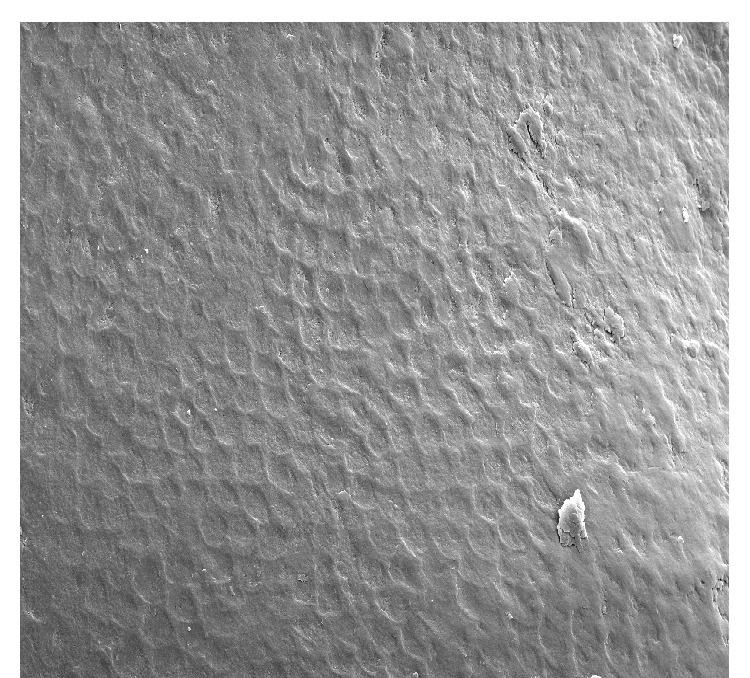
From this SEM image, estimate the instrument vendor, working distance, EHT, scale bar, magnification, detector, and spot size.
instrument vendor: FEI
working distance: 14.9 mm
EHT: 7 kV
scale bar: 20000 nm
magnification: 1 K X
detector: SE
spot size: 3